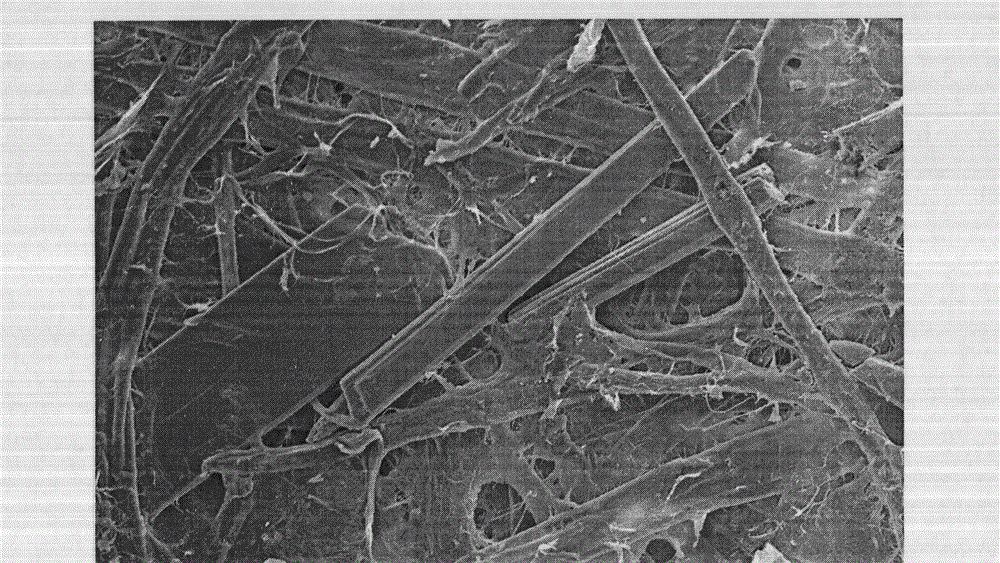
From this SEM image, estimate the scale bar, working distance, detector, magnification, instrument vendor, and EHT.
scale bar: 100000 nm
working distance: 10 mm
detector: SE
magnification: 0.3 K X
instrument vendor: Hitachi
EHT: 15 kV